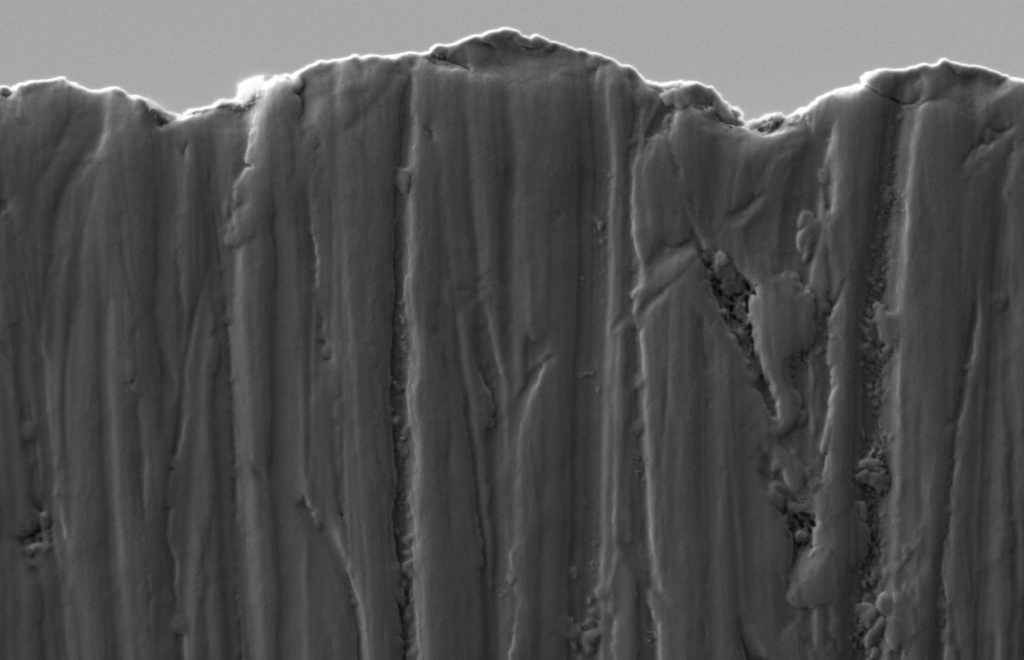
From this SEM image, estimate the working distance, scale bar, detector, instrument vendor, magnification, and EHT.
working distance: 5.1 mm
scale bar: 200 nm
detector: SE2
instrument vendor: Zeiss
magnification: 20 K X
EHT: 1 kV